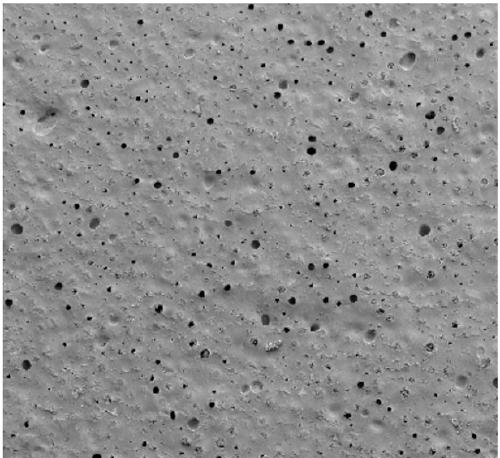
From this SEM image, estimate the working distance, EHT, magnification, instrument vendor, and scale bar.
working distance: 9.2 mm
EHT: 2 kV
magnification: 0.2 K X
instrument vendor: other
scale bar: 200000 nm